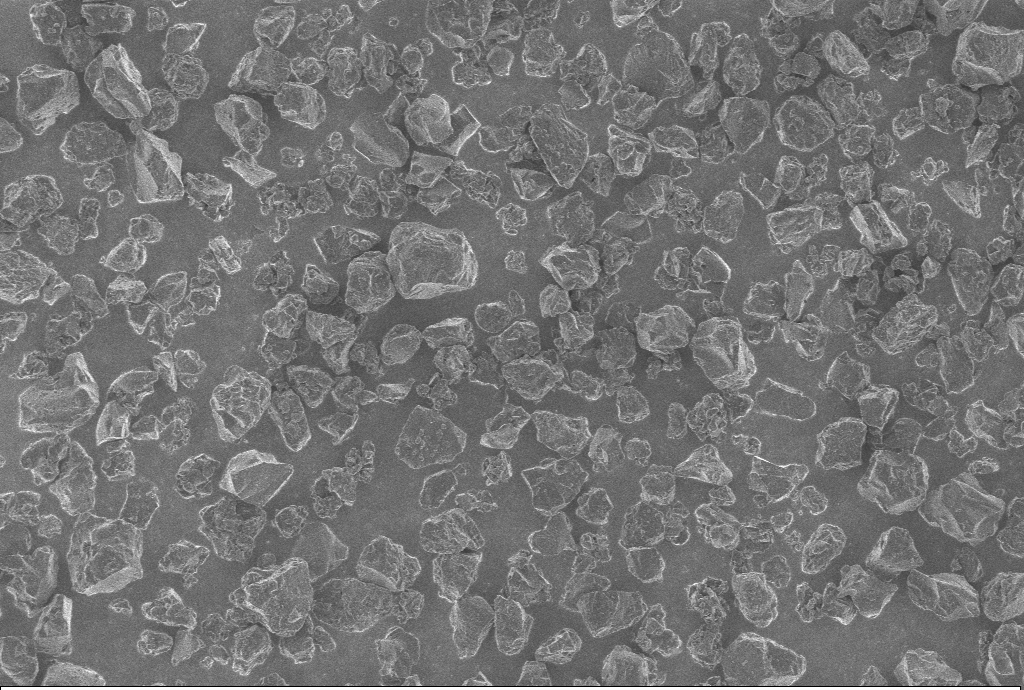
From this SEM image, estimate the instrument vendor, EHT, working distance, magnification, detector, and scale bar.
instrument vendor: Zeiss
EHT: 1 kV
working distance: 5.6 mm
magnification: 1 K X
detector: InLens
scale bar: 10000 nm